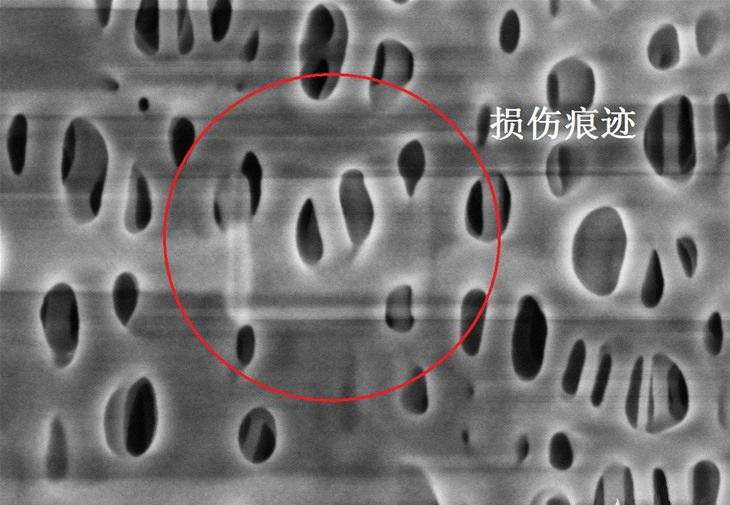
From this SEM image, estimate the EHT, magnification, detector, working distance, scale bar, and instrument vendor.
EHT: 1 kV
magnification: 100 K X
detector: SE(U,LA0)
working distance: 1.5 mm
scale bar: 500 nm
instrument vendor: Hitachi